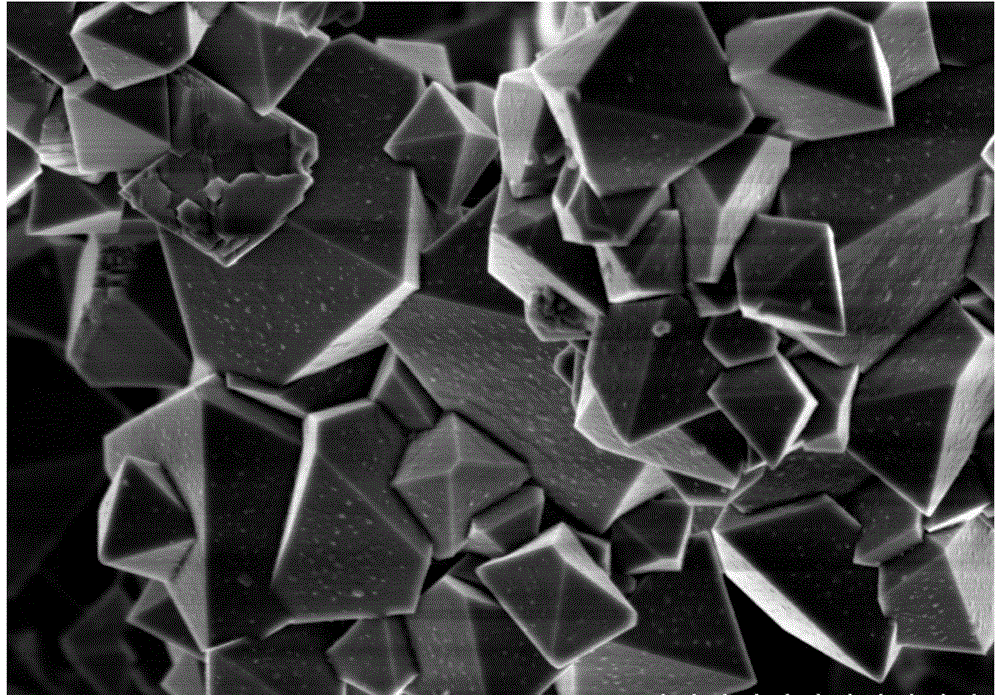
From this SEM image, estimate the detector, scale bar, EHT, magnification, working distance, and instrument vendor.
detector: SE(U)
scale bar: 2000 nm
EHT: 3 kV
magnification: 20 K X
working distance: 9.7 mm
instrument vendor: Hitachi